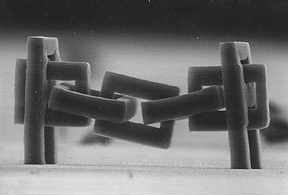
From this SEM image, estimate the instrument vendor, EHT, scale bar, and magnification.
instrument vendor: JEOL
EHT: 25 kV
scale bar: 100000 nm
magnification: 0.5 K X